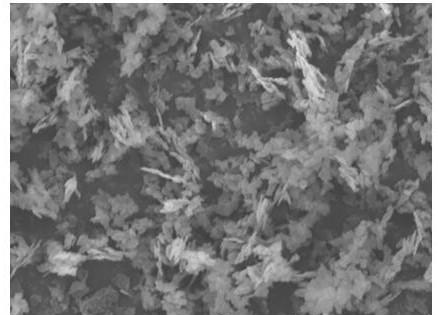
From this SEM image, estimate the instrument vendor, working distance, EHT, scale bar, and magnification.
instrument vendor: Tescan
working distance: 14.71 mm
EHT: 20 kV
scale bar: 5000 nm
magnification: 5 K X